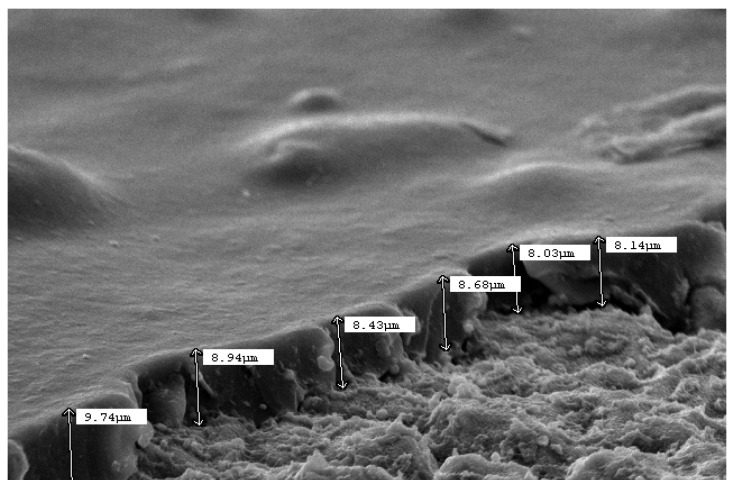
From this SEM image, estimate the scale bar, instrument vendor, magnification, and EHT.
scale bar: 10000 nm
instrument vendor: JEOL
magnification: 1.5 K X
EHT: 15 kV